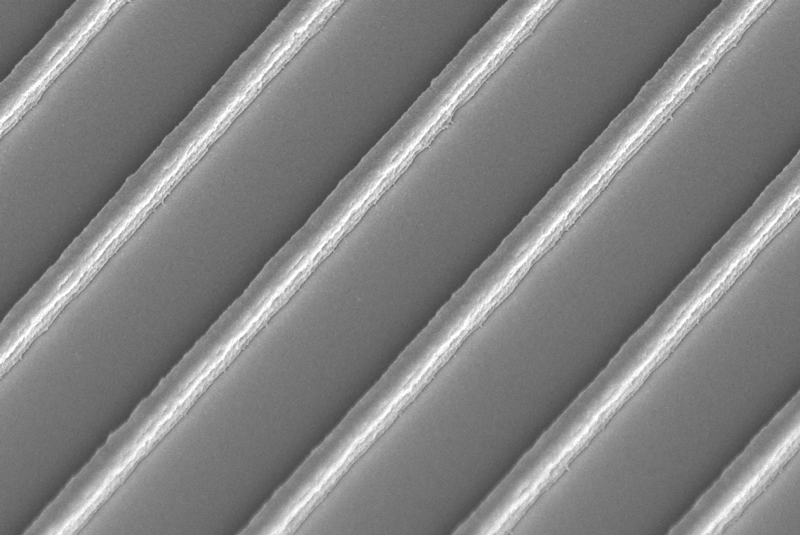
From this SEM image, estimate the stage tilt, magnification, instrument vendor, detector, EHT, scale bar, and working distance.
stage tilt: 45°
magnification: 13.21 K X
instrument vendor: Zeiss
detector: InLens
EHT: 3 kV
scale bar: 1000 nm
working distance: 7 mm